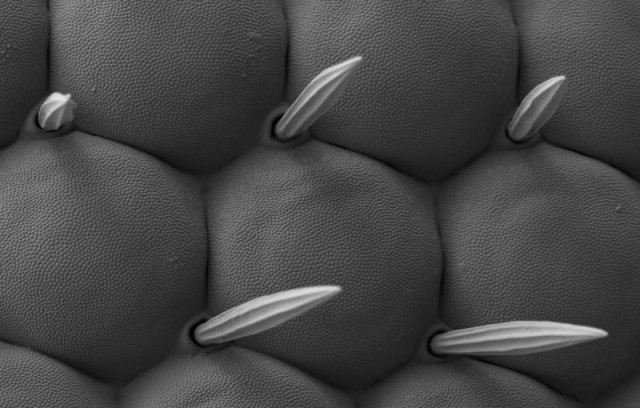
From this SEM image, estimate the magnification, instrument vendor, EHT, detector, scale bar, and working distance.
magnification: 3 K X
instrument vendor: Zeiss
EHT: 1 kV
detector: SE2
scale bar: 2000 nm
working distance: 4.2 mm